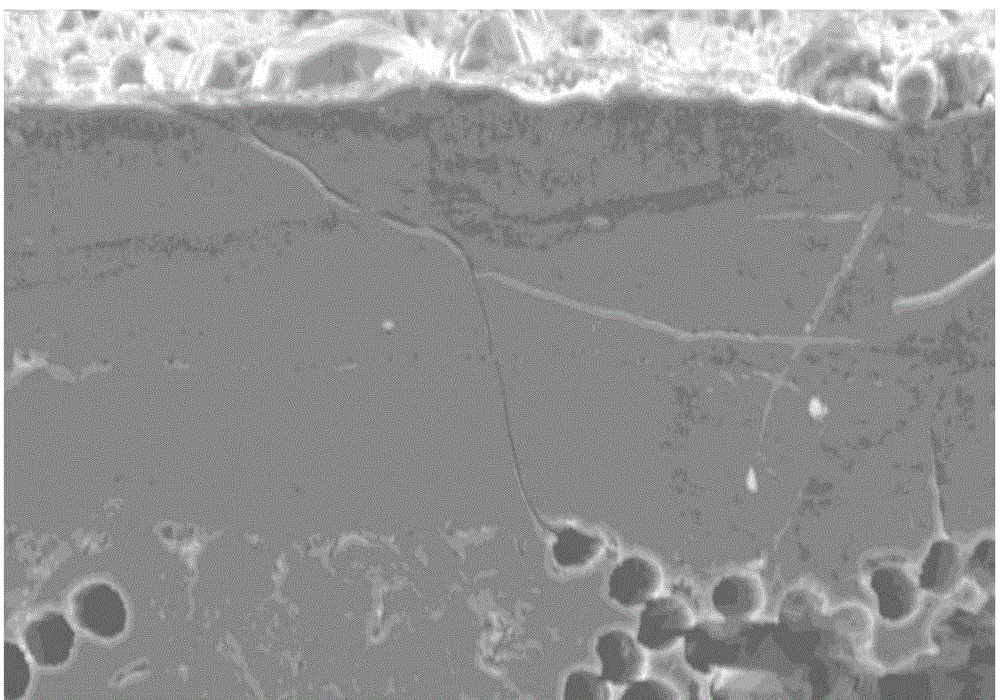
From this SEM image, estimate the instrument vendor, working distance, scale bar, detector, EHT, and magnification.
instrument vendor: Hitachi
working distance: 11.1 mm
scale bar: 50000 nm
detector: SE(M)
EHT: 15 kV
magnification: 1 K X